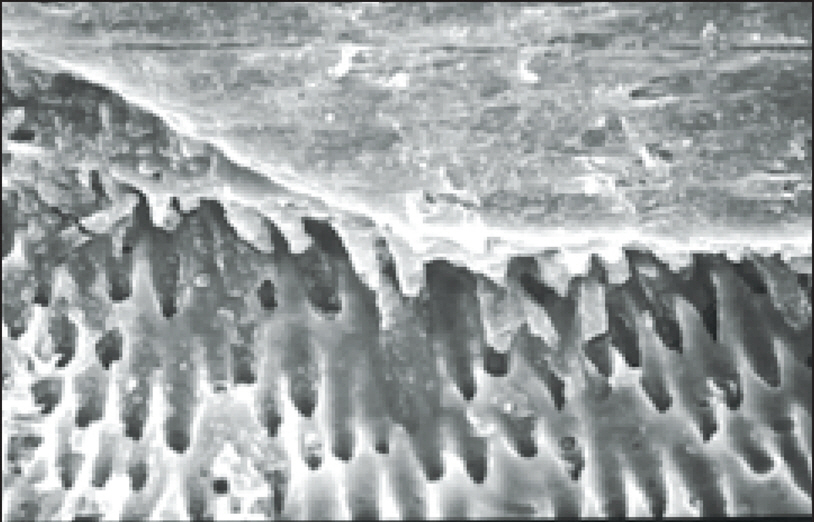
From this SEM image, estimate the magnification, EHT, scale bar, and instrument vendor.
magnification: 1 K X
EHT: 20 kV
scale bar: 50000 nm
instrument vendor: Hitachi